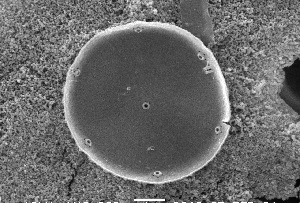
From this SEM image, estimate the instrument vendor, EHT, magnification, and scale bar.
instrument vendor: JEOL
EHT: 10 kV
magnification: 13 K X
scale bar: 1000 nm